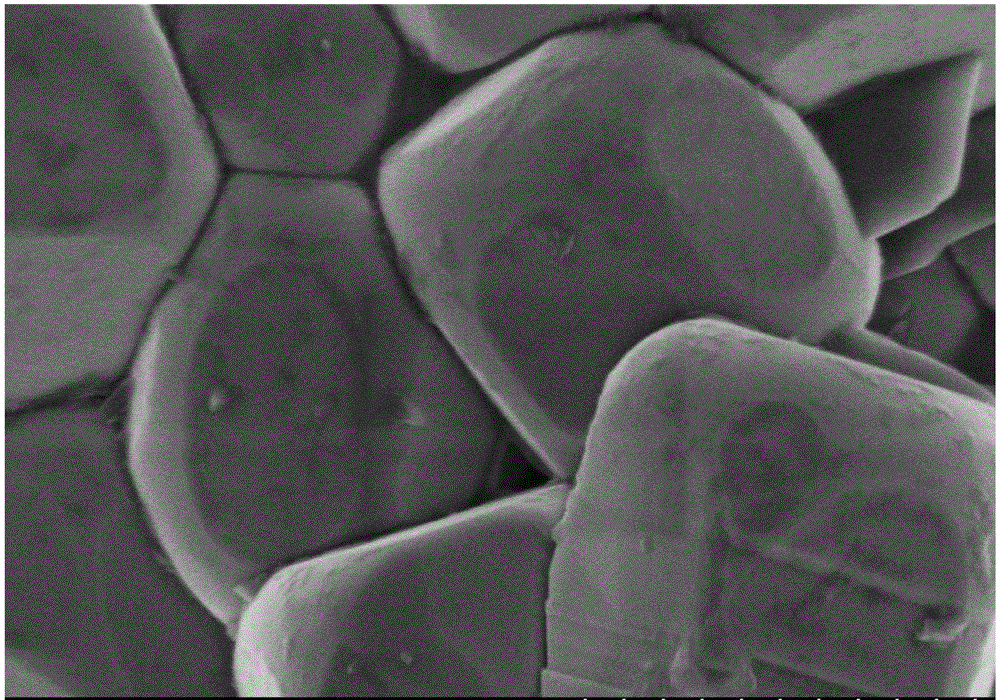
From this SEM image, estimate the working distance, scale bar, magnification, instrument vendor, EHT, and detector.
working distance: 7.9 mm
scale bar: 1000 nm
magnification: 50 K X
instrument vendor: Hitachi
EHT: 3 kV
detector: SE(M)